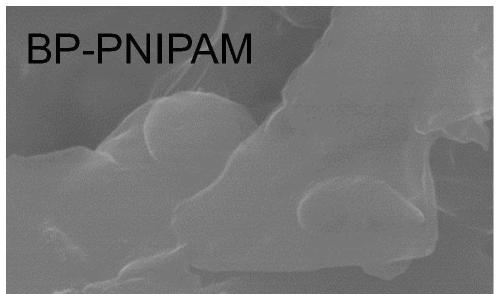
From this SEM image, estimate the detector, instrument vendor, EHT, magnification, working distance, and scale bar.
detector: SE(UL)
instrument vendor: Hitachi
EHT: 15 kV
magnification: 60 K X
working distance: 8.5 mm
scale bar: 500 nm